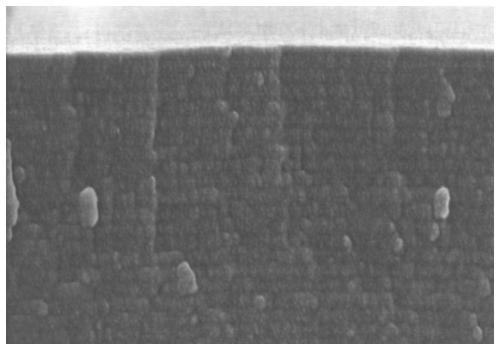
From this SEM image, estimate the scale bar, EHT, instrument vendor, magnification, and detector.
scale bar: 500 nm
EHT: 8 kV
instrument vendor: Hitachi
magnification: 70 K X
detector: SE(U)